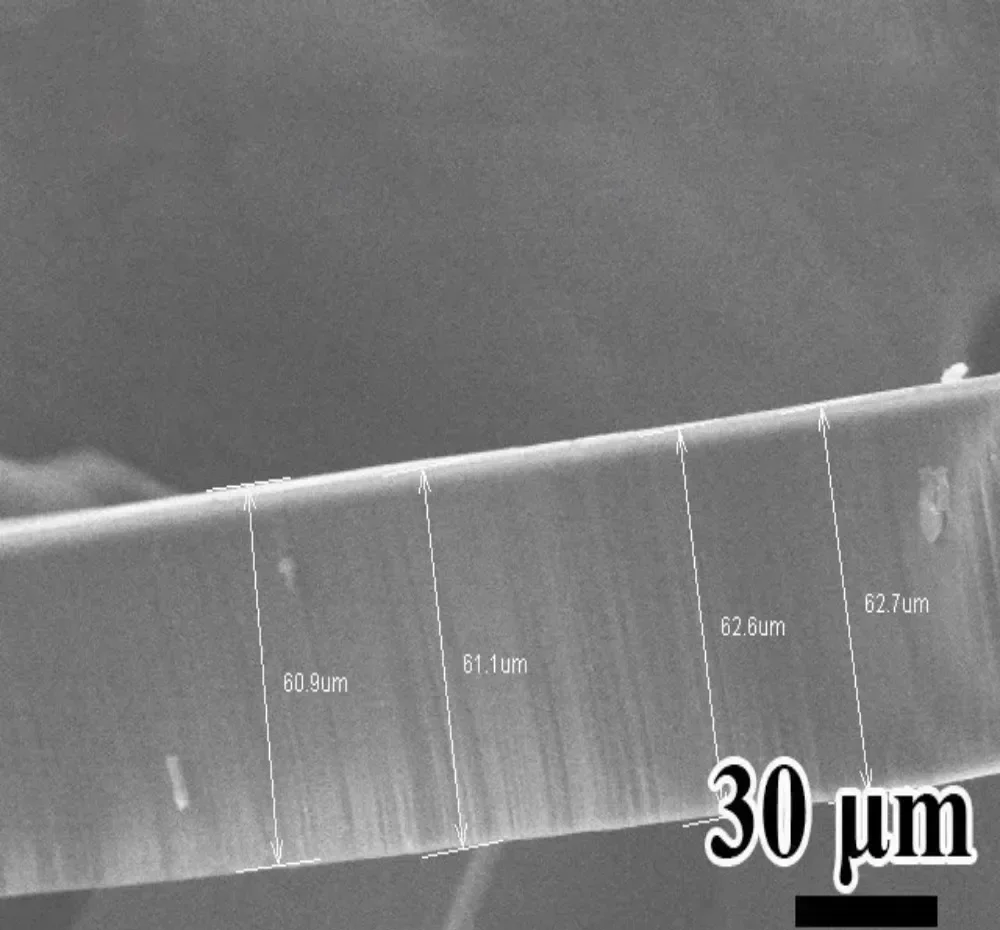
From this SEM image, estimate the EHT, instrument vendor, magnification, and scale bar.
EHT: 20 kV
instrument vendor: Hitachi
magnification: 0.6 K X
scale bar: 50000 nm